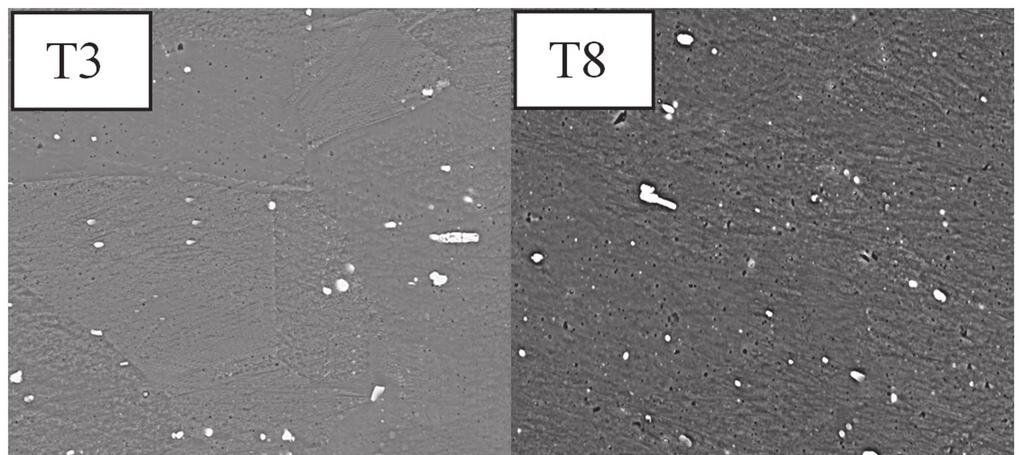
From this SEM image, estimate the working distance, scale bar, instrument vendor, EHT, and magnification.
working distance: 15 mm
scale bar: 20000 nm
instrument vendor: other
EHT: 15 kV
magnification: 1 K X